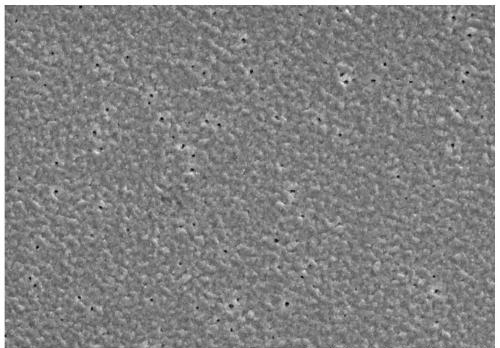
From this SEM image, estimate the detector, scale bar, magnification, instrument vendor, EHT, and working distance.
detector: SE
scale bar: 10000 nm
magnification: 5 K X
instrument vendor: Hitachi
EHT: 15 kV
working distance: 10 mm